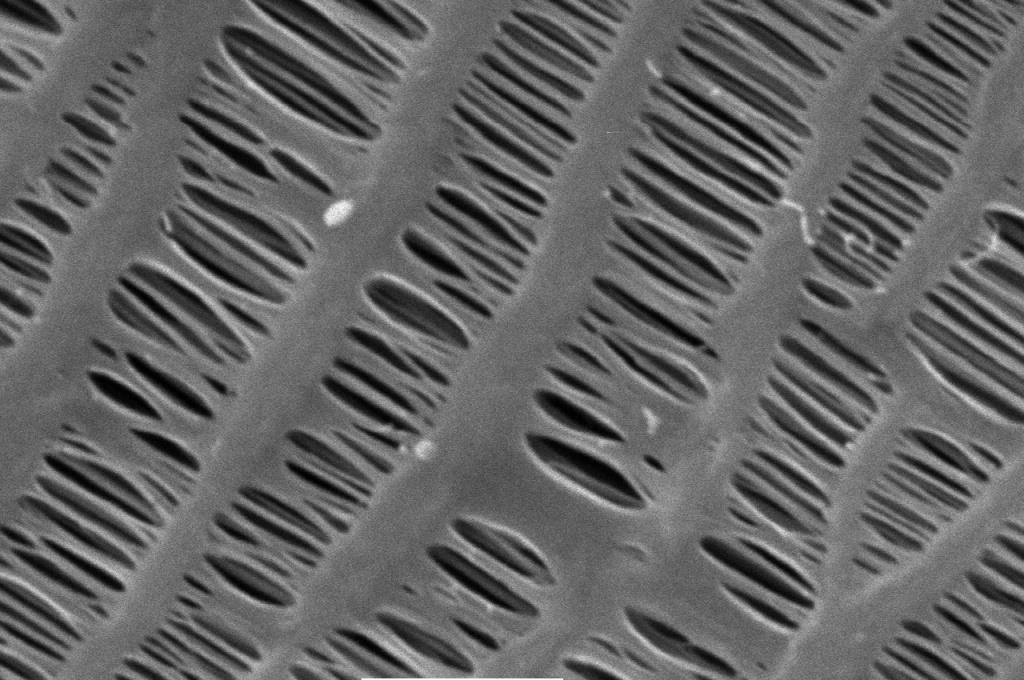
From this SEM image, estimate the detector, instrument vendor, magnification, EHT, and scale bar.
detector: SEI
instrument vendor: JEOL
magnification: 25 K X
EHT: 5 kV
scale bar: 1000 nm